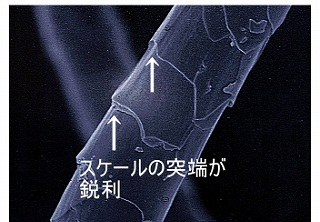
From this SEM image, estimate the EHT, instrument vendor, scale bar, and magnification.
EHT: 5 kV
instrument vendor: JEOL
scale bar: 10000 nm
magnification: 2 K X